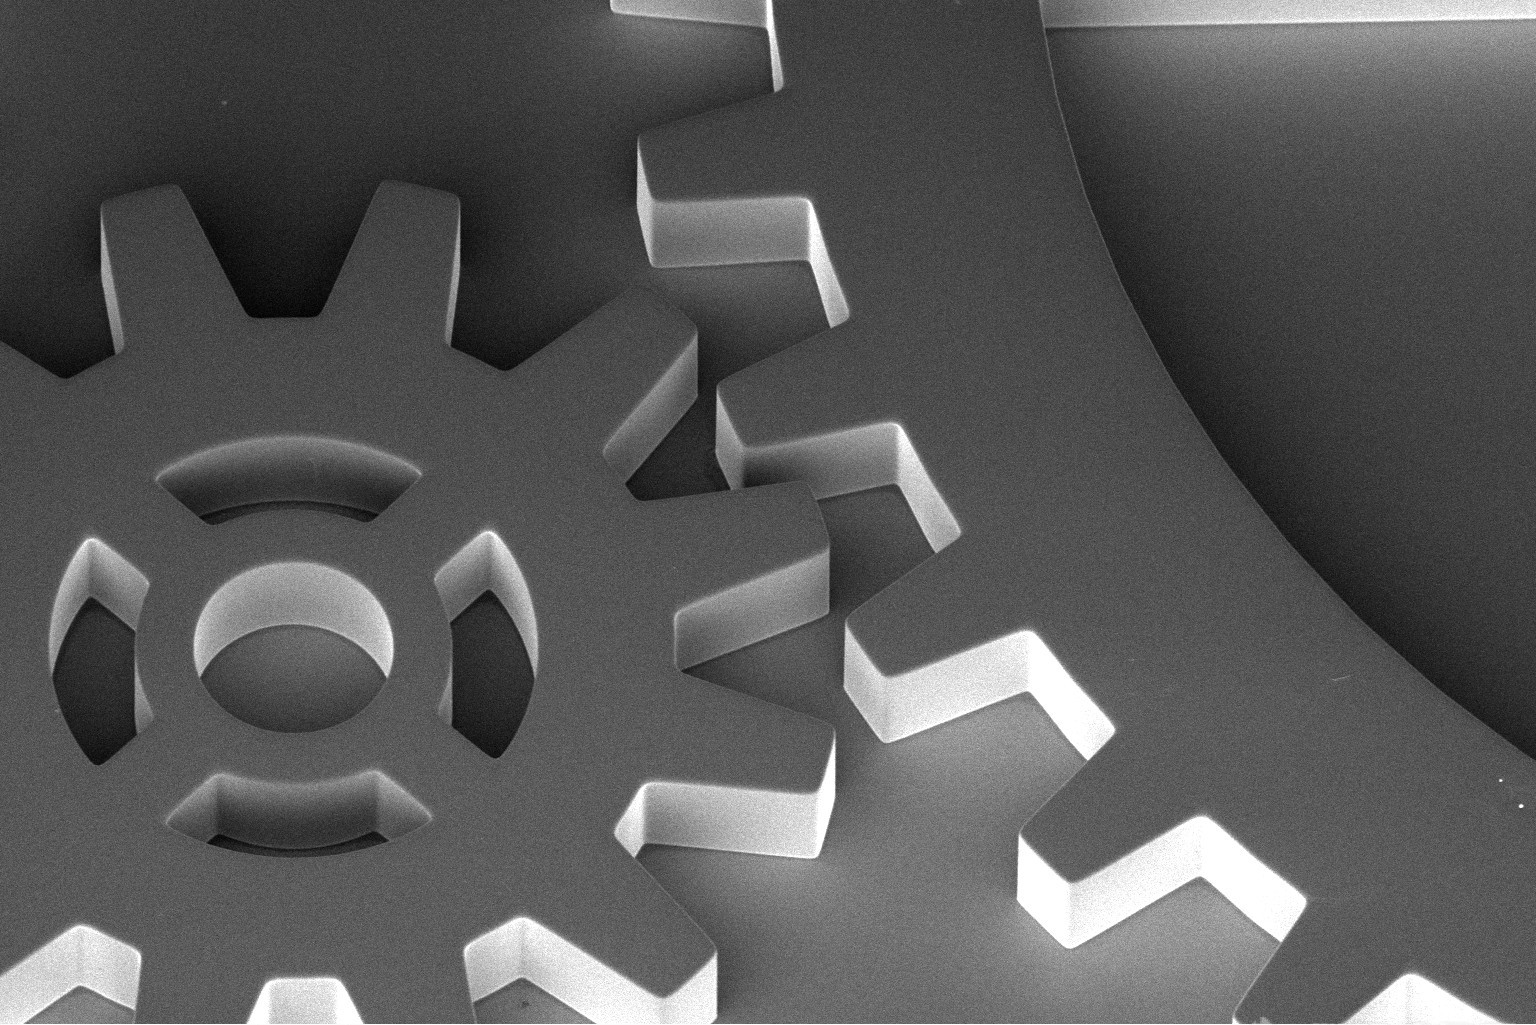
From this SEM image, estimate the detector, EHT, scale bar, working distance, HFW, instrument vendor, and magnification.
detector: TLD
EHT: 2 kV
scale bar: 100000 nm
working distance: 4 mm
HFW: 592 µm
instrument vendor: FEI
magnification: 0.35 K X